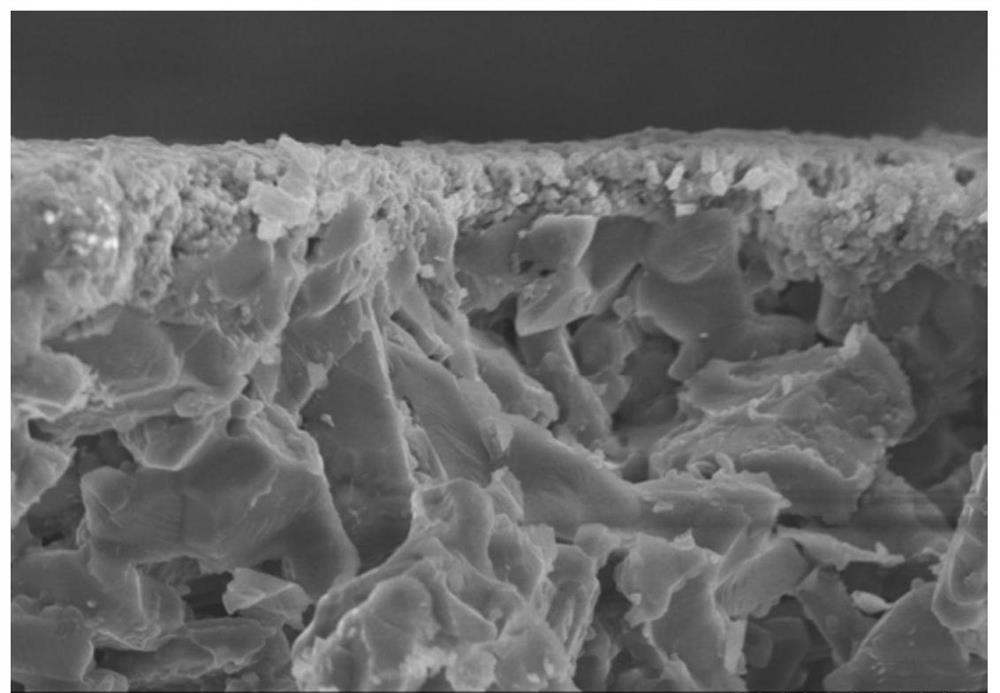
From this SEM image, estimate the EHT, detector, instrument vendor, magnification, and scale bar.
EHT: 8 kV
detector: SE(M)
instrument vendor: Hitachi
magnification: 6 K X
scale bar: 5000 nm